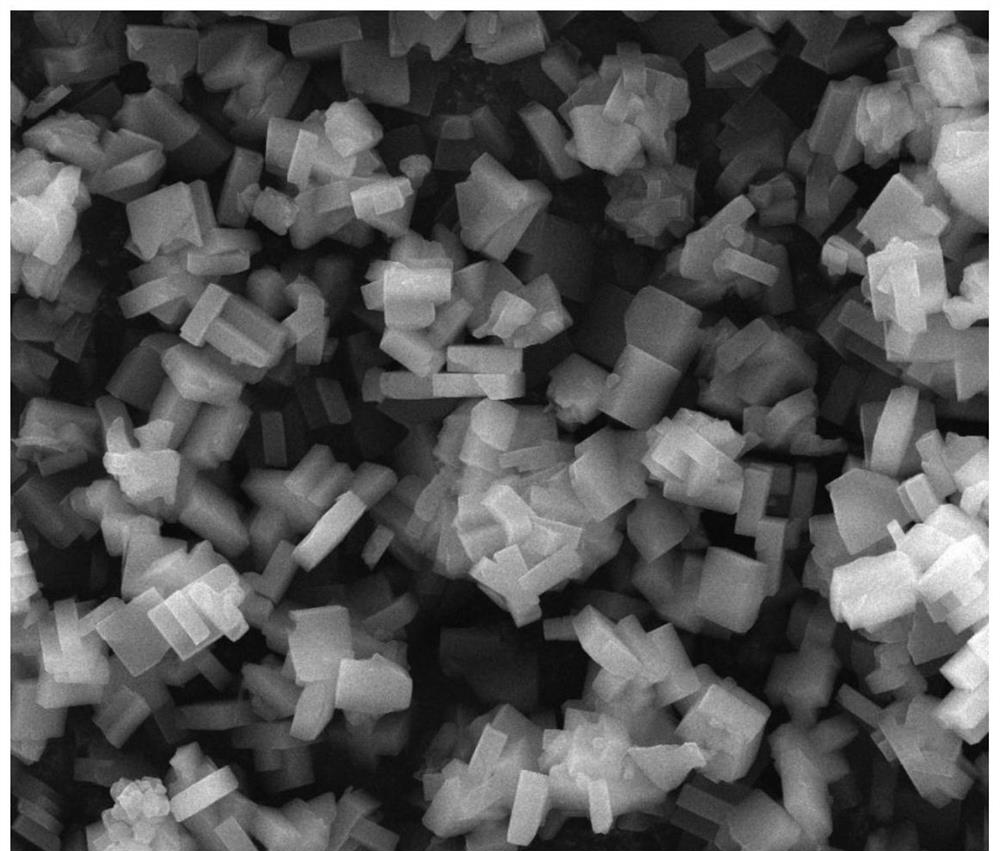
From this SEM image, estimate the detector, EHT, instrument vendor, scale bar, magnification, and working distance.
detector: ETD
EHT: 20 kV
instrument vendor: FEI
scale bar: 10000 nm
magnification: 10 K X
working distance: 11.6 mm